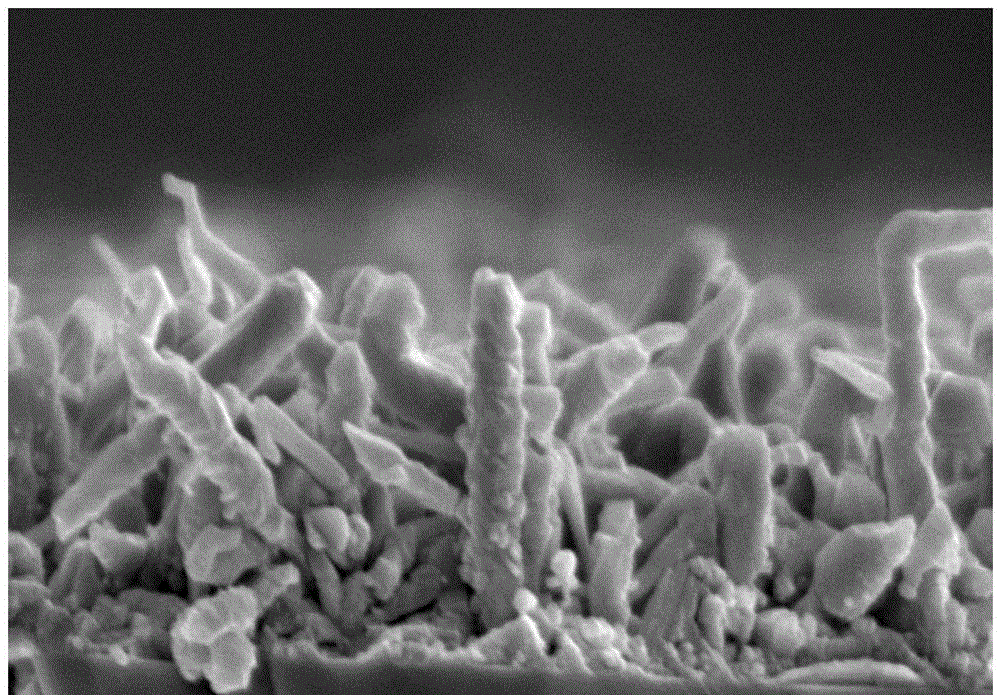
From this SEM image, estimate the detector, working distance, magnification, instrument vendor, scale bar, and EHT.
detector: SE(M)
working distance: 5.5 mm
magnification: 40 K X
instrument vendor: Hitachi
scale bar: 1000 nm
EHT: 5 kV